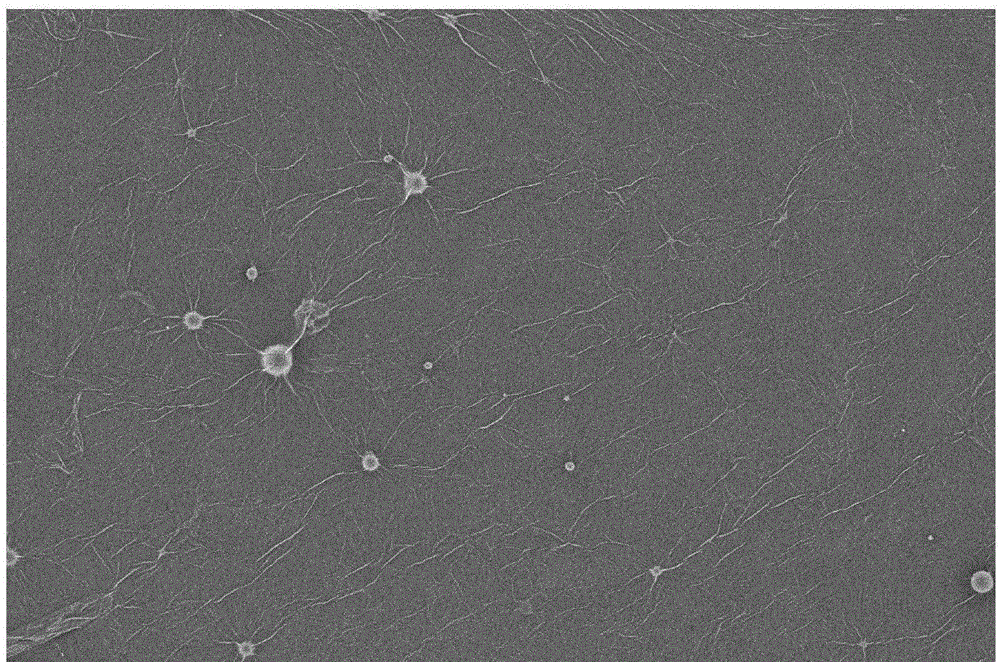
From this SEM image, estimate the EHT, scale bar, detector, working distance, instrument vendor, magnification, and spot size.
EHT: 1 kV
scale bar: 5000 nm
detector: CBS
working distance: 5.8 mm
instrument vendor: FEI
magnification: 10 K X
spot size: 3